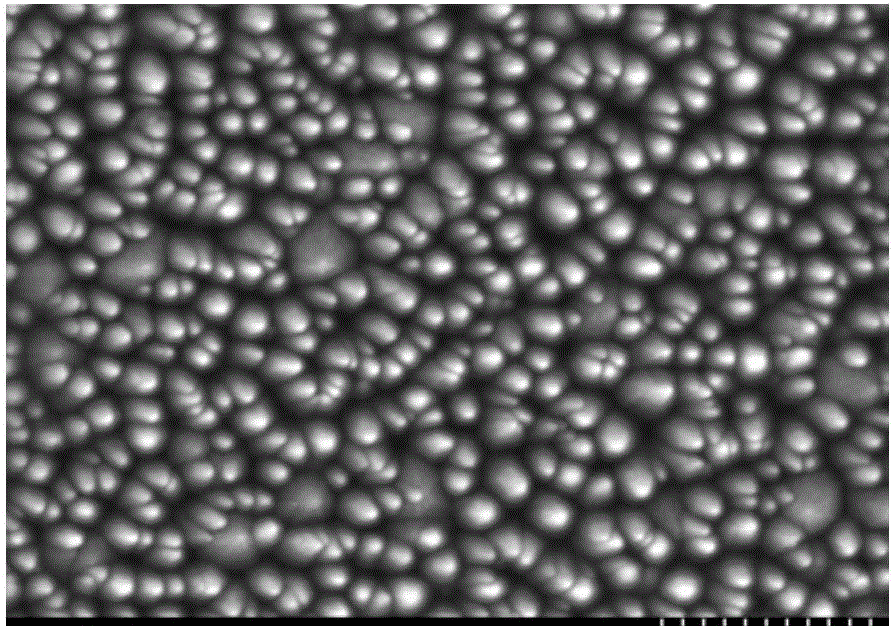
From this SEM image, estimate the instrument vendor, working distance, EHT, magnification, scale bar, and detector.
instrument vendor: Hitachi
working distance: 8.6 mm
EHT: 5 kV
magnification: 30 K X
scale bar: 1000 nm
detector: SE(M)